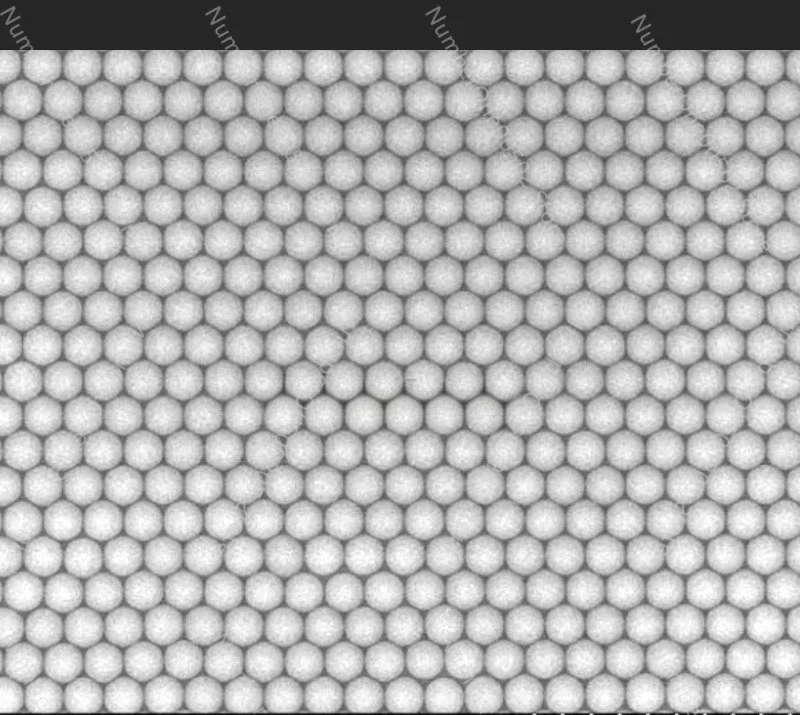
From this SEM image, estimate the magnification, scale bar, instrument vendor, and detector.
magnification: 3.5 K X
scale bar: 10000 nm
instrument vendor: Hitachi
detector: SE